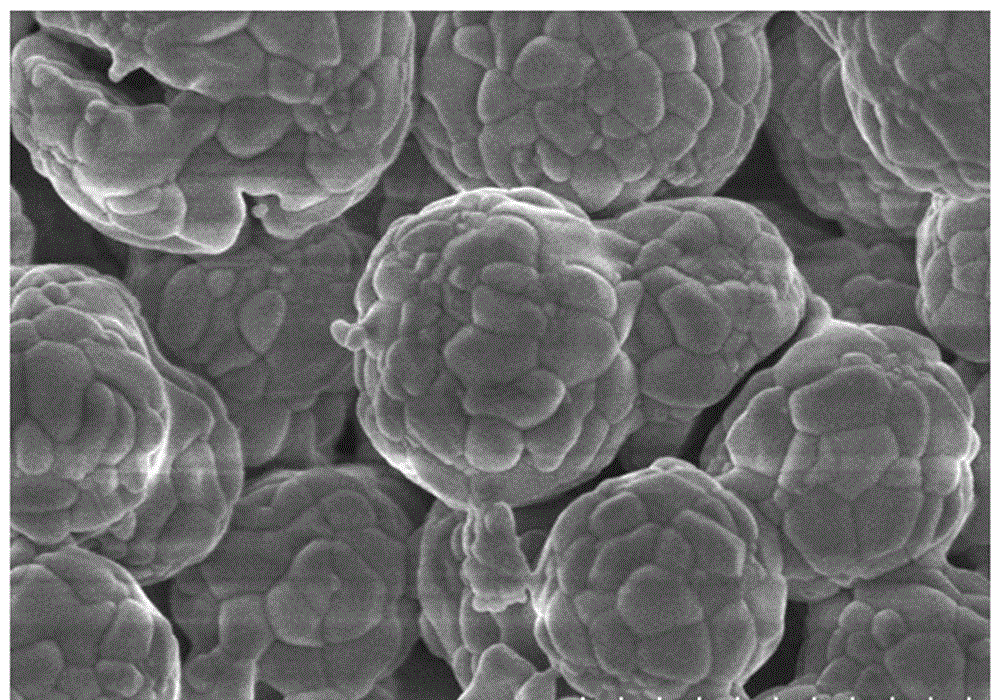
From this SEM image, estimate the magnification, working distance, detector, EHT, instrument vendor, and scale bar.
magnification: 25 K X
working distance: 3.8 mm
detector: SE(M)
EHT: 3 kV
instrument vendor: Hitachi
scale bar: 2000 nm